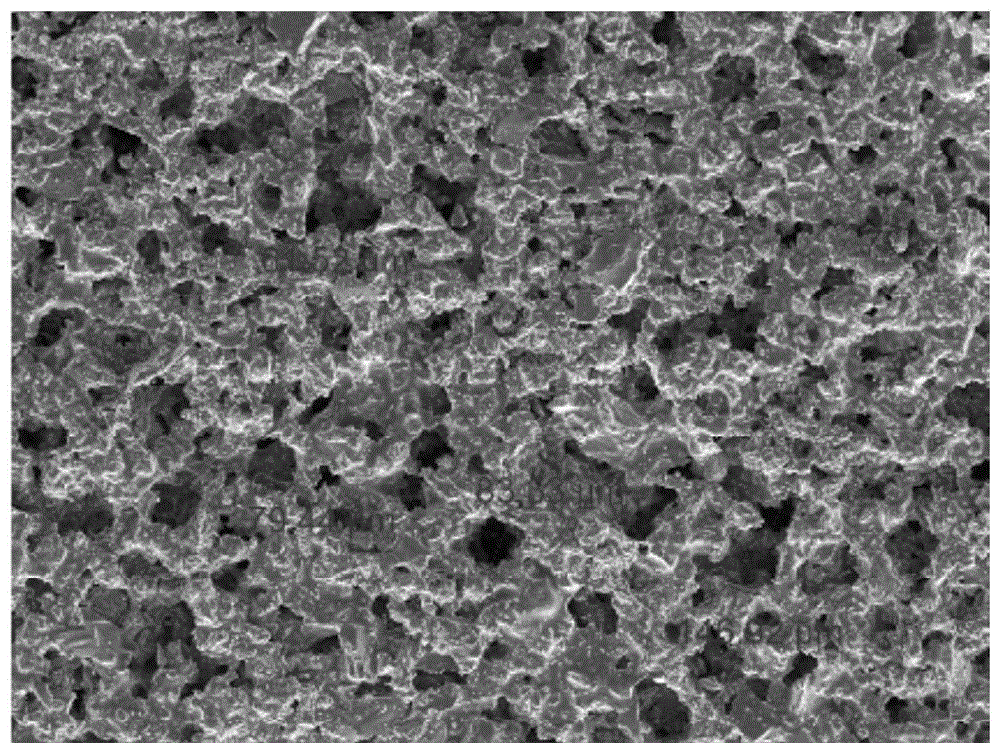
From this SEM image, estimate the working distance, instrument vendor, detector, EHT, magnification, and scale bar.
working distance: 14.5 mm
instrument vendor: Tescan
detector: SE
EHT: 10 kV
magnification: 0.2 K X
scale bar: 200000 nm